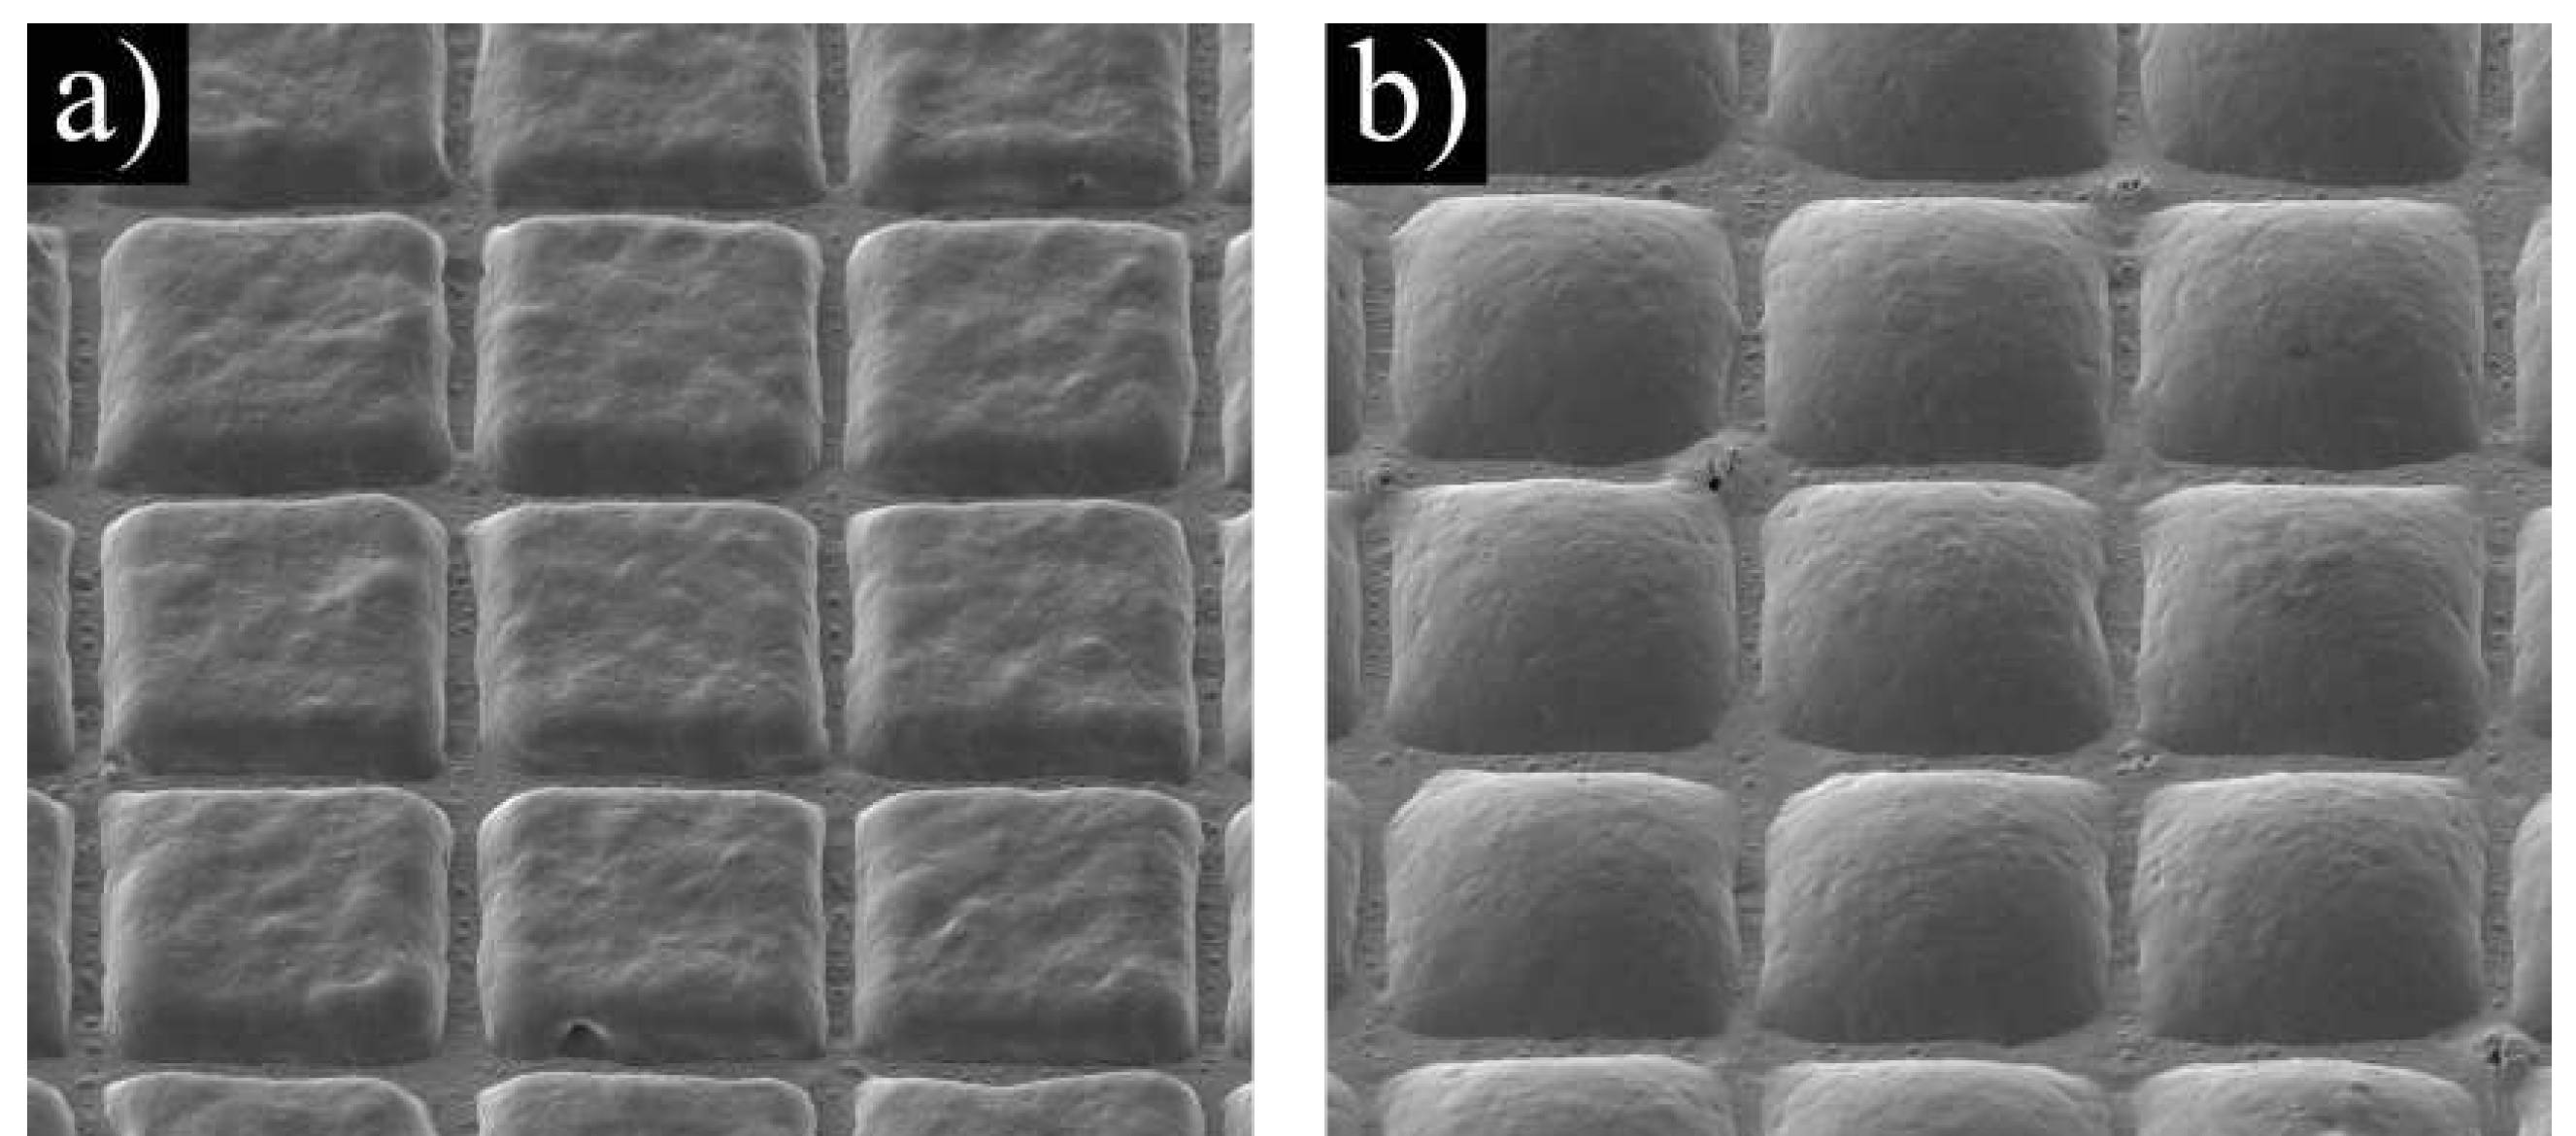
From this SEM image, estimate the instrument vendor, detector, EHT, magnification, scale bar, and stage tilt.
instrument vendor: Tescan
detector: SE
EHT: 5 kV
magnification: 0.8 K X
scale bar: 50000 nm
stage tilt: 35°